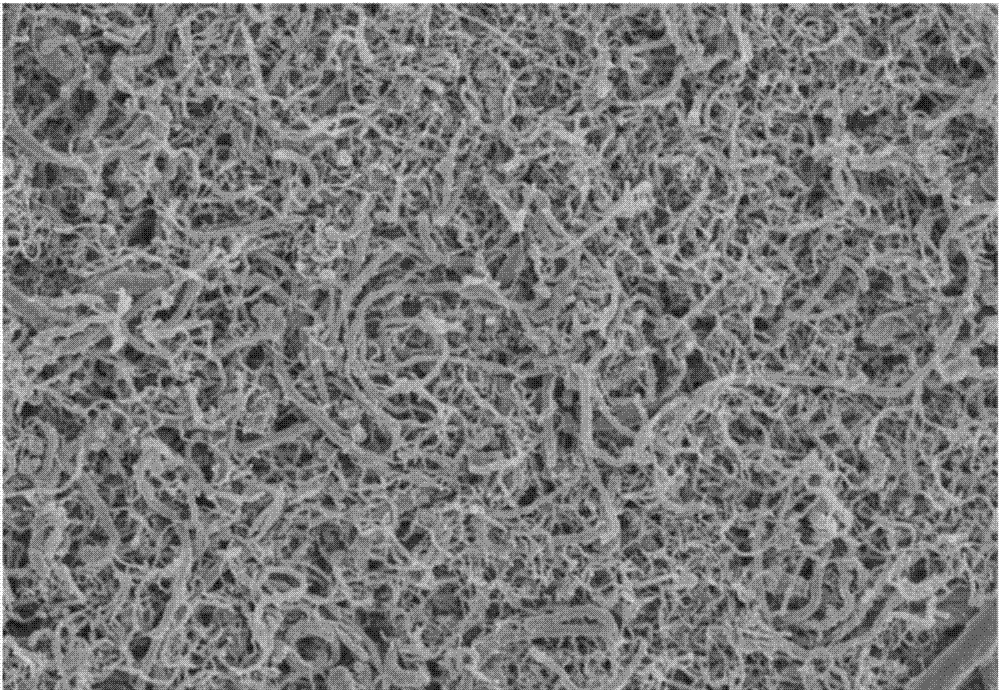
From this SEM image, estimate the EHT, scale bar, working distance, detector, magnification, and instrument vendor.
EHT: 3 kV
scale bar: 5000 nm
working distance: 9.8 mm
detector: SE(UL)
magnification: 10 K X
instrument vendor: Hitachi